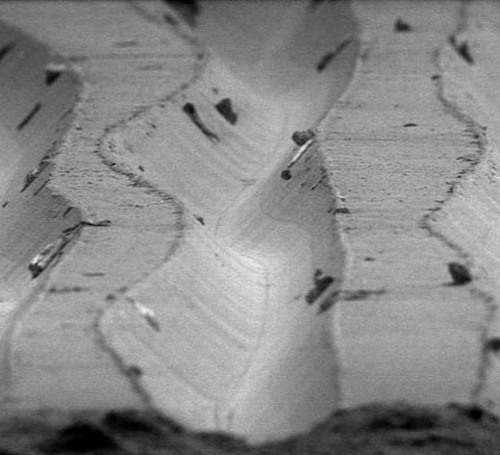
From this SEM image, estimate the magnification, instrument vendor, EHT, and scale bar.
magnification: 0.48 K X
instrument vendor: JEOL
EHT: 5 kV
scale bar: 50000 nm